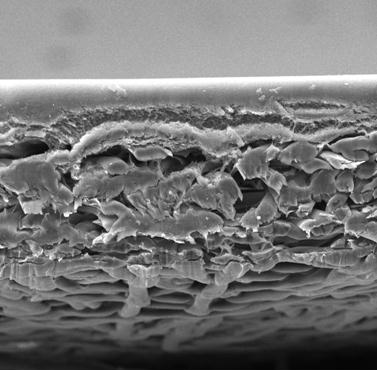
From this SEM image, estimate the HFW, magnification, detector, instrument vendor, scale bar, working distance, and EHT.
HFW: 433 µm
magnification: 0.5 K X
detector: SE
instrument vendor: Tescan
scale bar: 100000 nm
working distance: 15.61 mm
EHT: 20 kV